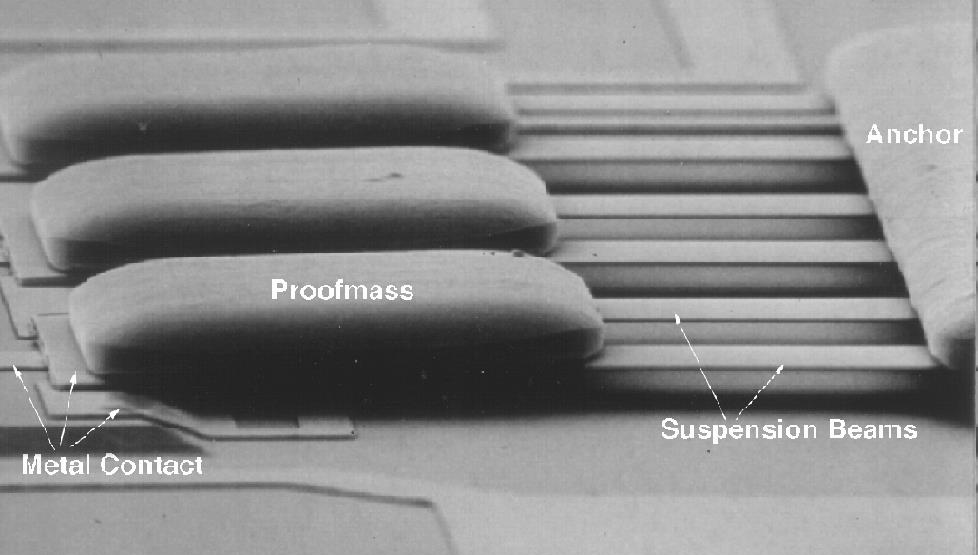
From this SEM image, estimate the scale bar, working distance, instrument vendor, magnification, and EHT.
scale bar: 10000 nm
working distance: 26 mm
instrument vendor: JEOL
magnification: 0.65 K X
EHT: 10 kV